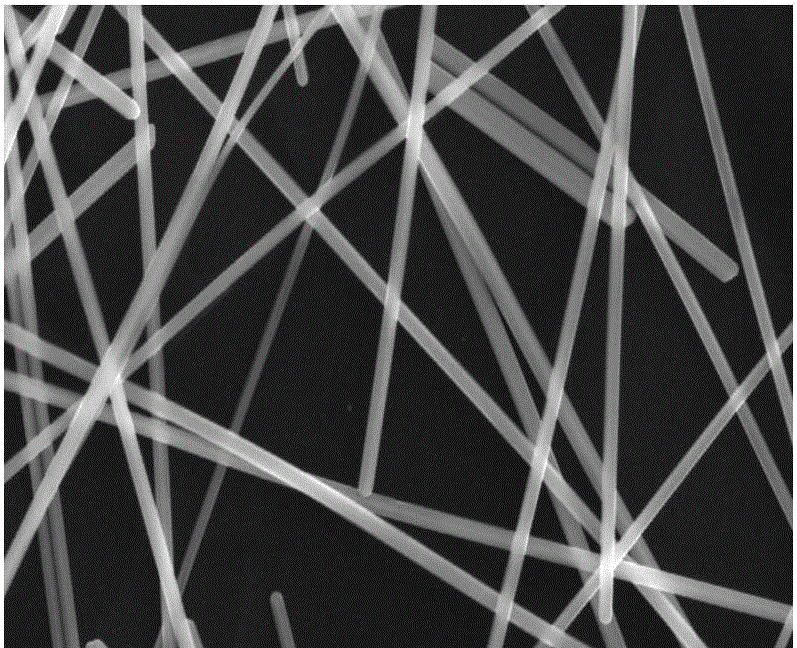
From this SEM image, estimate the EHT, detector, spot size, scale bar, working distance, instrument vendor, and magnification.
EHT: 20 kV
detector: SE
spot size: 4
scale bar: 2000 nm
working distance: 6.7 mm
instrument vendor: FEI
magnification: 16 K X